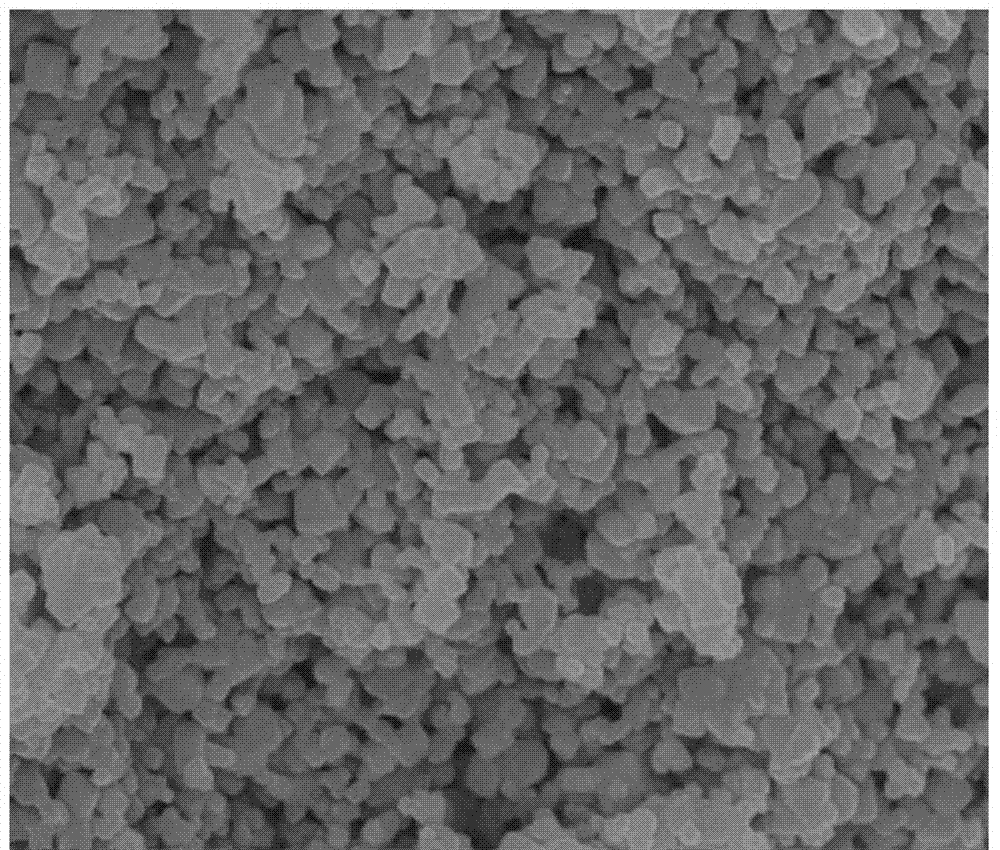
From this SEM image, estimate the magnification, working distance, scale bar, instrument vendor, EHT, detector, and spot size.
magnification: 50 K X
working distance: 5.2 mm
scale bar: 1000 nm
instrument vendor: FEI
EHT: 10 kV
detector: TLD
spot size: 3